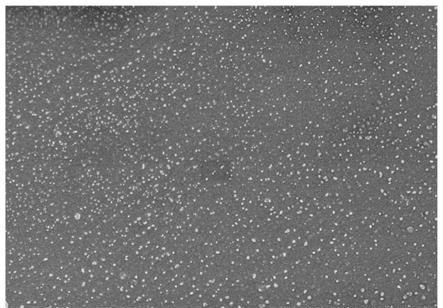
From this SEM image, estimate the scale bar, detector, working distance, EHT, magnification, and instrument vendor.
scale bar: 20000 nm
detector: SE(M)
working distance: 8 mm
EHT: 5 kV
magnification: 2 K X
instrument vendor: Hitachi